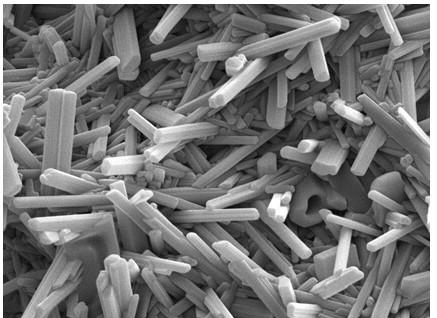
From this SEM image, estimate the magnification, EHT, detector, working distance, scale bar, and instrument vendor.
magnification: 20 K X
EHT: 10 kV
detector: ETD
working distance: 10.7 mm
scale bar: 5000 nm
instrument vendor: FEI